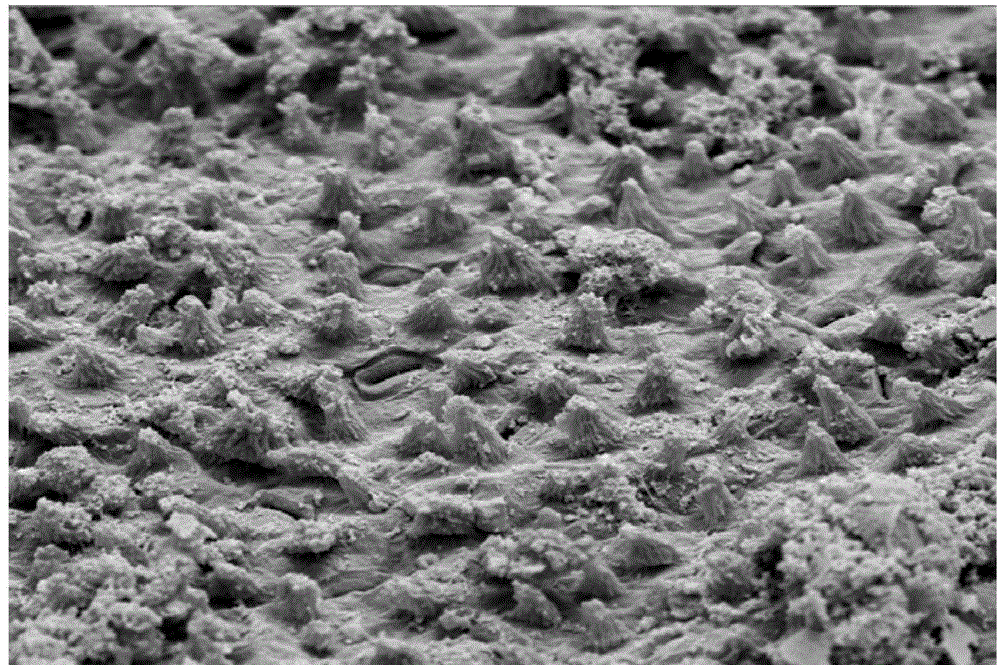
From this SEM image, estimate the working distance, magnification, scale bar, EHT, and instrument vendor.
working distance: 24 mm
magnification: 1 K X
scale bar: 10000 nm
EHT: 15 kV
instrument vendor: JEOL